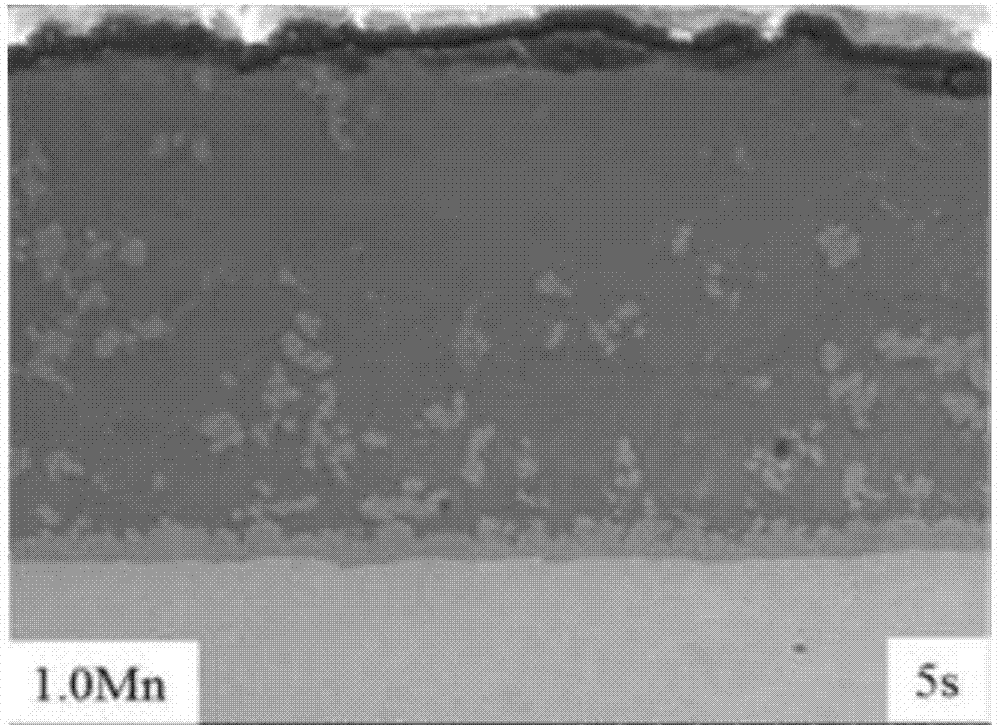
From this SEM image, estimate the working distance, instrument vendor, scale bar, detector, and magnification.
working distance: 14.9 mm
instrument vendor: Hitachi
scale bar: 50000 nm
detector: SE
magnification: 1 K X